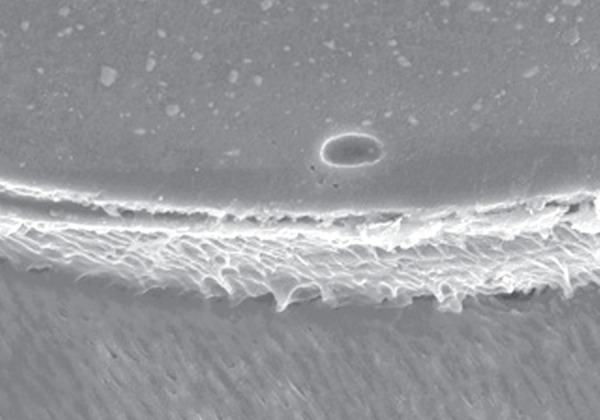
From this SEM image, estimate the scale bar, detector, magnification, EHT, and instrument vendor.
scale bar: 50000 nm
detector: SEI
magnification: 0.5 K X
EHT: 25 kV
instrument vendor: JEOL